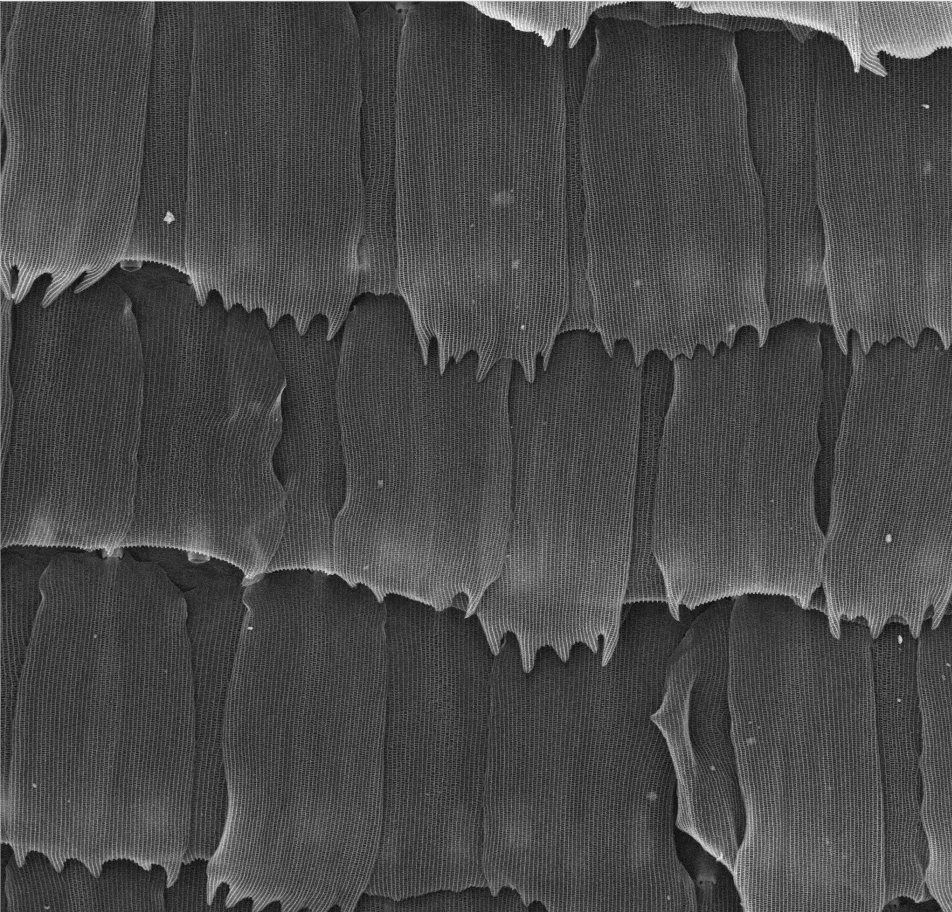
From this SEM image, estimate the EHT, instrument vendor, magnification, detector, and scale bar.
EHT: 15 kV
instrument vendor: other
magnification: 1 K X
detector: SE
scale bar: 50000 nm